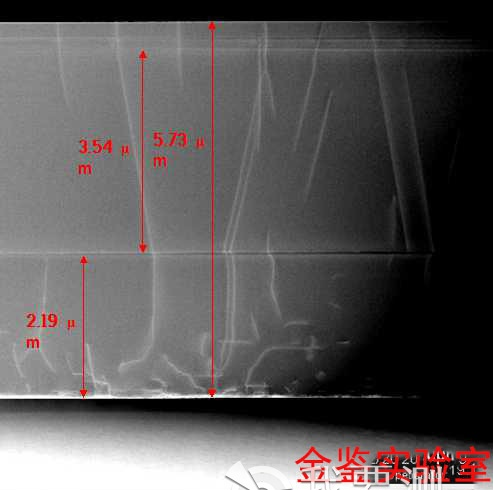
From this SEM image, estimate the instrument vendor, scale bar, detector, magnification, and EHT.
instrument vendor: JEOL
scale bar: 2000 nm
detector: STEM BF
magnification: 20 K X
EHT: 200 kV